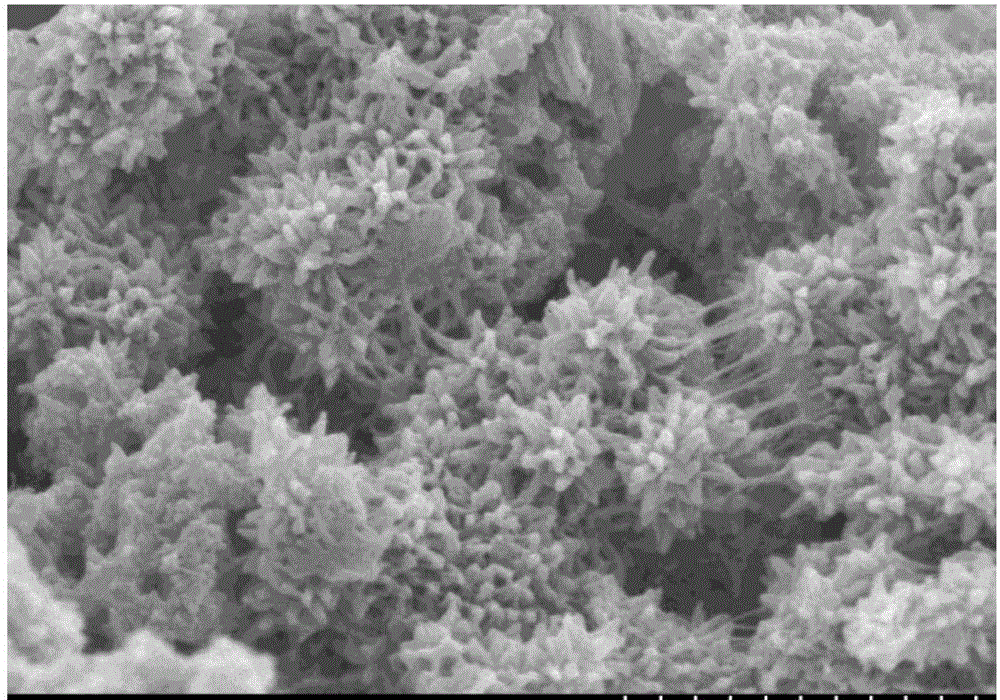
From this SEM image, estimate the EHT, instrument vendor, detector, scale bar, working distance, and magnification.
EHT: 3 kV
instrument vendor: Hitachi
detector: SE(M)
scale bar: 1000 nm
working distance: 9.6 mm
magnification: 45 K X